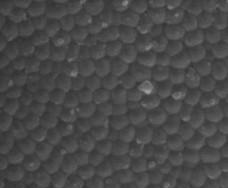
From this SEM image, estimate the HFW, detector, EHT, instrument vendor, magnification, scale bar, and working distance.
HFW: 1.5 µm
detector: LFD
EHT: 10 kV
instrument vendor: FEI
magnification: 200 K X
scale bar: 500 nm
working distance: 5.8 mm